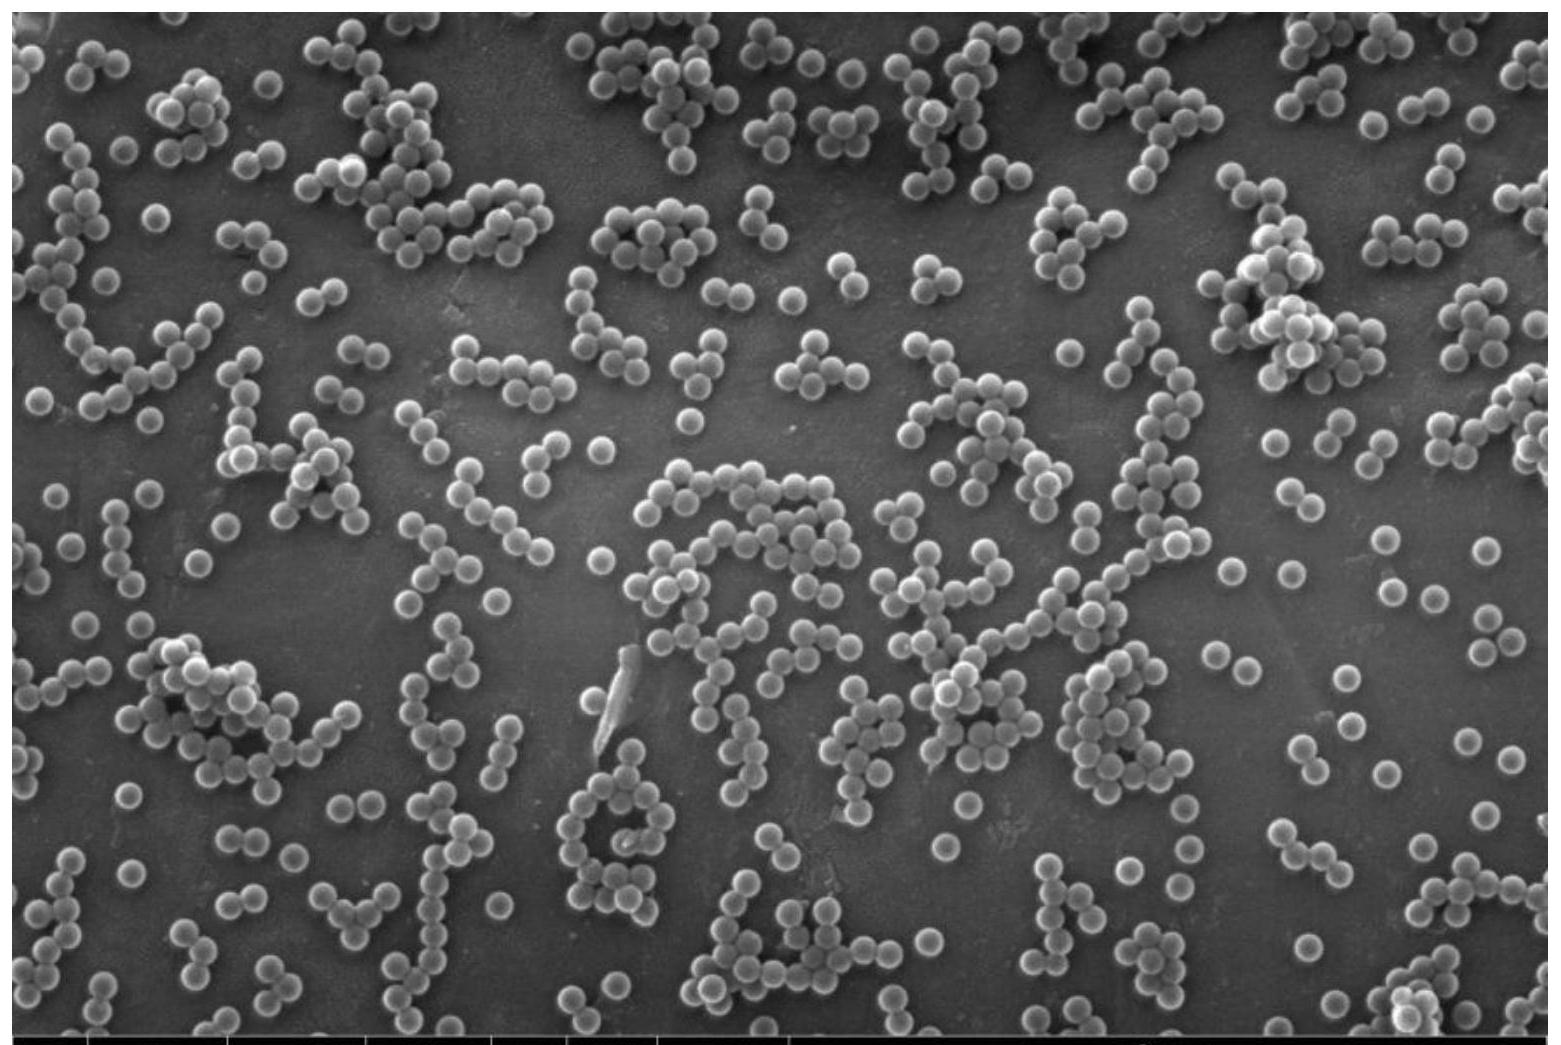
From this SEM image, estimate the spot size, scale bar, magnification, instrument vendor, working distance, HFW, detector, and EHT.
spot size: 5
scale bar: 3000 nm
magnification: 50 K X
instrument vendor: FEI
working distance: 9.7 mm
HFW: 8.29 µm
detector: SE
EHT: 30 kV